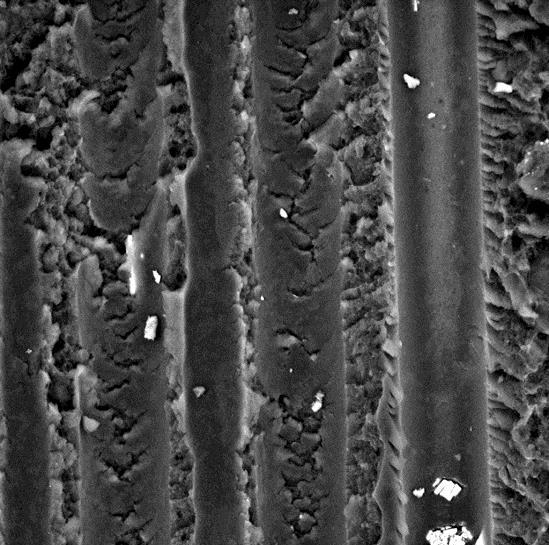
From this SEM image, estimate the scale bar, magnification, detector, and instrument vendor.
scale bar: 10000 nm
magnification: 5 K X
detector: BSD Full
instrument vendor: other